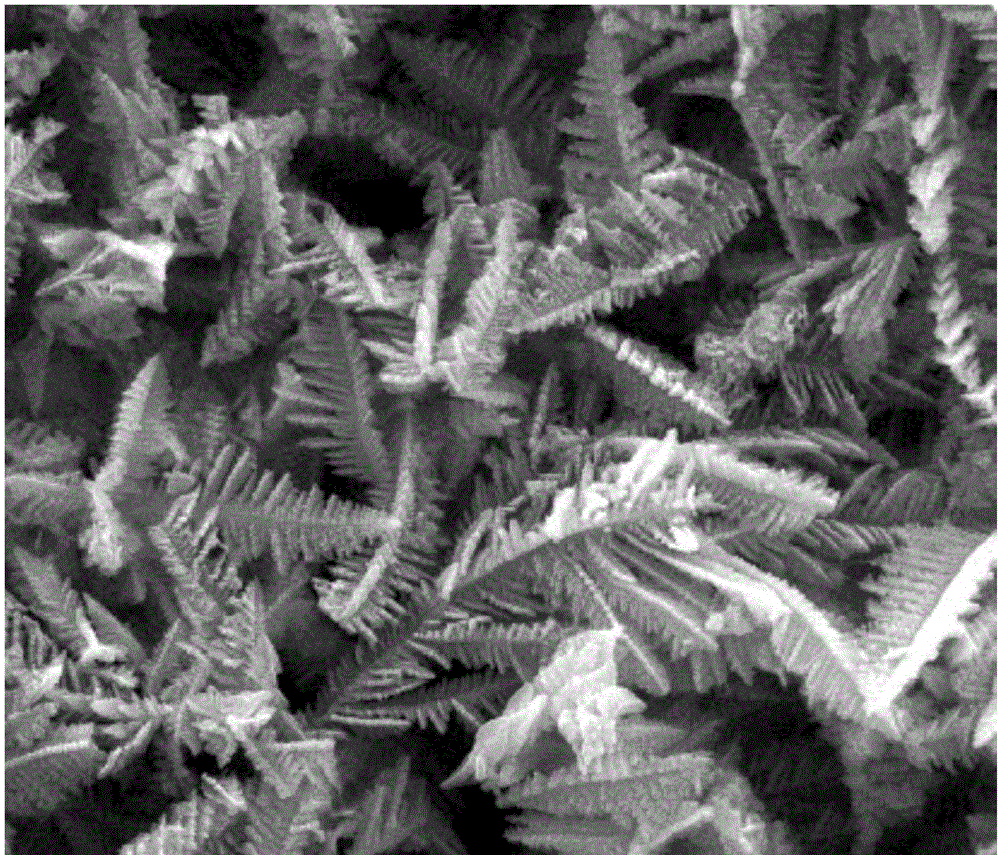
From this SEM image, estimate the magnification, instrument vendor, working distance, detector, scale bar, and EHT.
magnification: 20 K X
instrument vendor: FEI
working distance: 14.2 mm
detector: SE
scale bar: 5000 nm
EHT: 30 kV